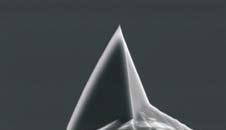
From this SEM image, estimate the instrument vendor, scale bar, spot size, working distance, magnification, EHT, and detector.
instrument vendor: FEI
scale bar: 5000 nm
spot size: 3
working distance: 21.8 mm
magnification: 5 K X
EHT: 10 kV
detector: SE